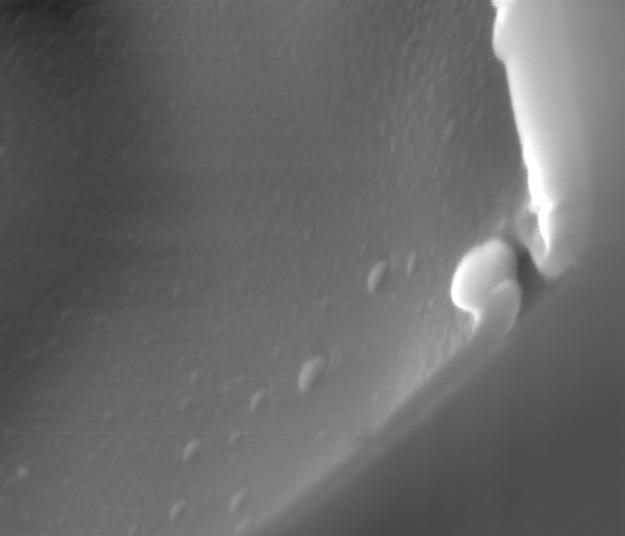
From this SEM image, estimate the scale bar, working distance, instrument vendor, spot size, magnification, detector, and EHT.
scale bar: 1000 nm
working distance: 10 mm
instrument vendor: FEI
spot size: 1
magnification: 59.996 K X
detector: ETD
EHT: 15 kV